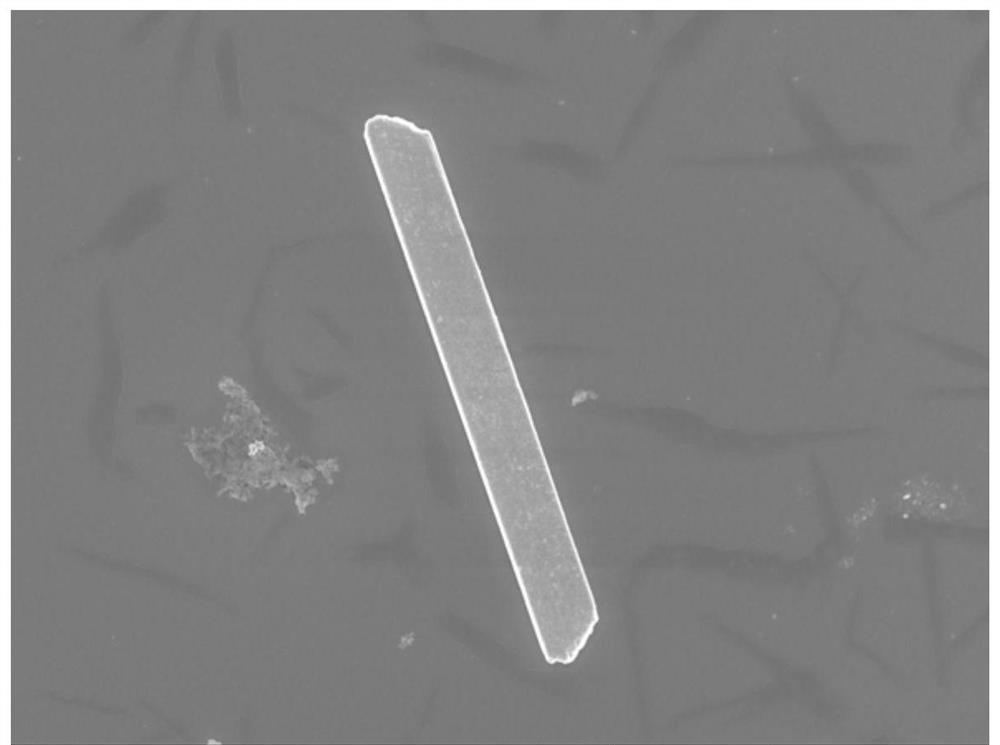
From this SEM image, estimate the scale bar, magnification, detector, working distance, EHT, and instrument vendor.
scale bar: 10000 nm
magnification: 2 K X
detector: SEI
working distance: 6.2 mm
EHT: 6 kV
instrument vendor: JEOL